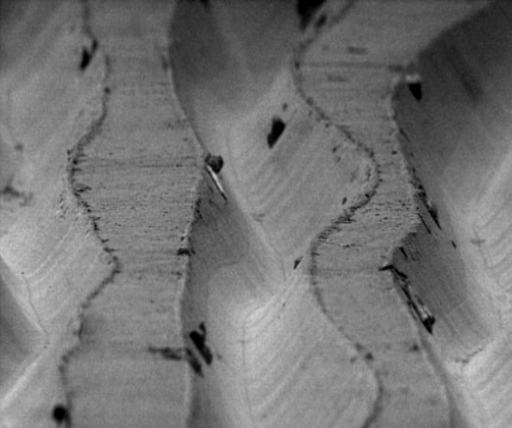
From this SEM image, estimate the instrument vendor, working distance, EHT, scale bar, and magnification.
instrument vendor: other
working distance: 27 mm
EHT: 5 kV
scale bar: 50000 nm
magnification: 0.5 K X